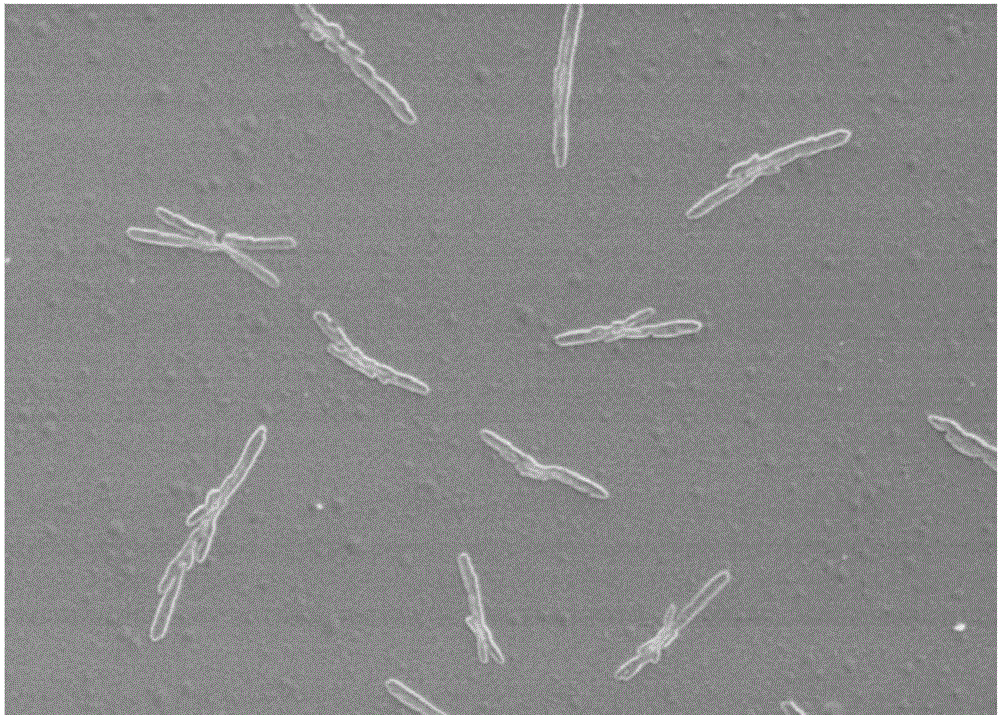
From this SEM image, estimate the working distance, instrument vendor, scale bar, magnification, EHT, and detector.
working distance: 9.1 mm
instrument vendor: Hitachi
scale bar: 20000 nm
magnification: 2 K X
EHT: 3 kV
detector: SE(M)